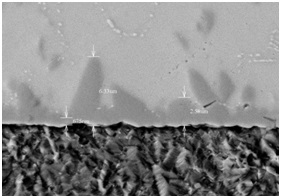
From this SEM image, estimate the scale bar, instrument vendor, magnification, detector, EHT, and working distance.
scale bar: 10000 nm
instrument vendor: Hitachi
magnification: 5 K X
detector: BSE3D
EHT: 15 kV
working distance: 8.9 mm